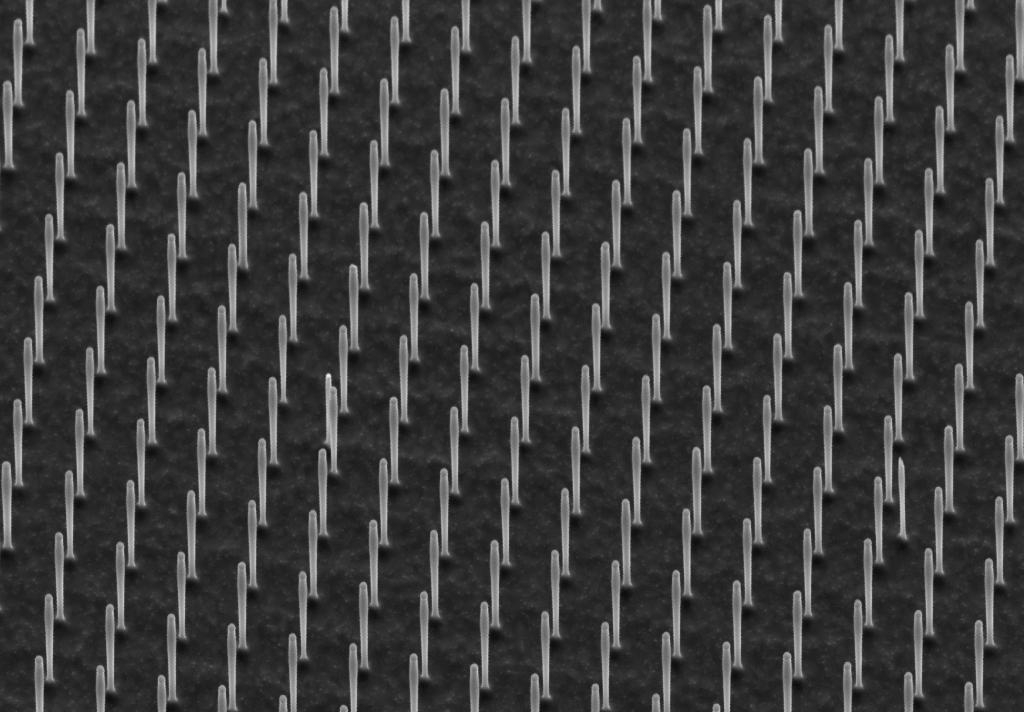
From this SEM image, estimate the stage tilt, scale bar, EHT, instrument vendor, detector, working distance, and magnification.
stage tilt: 45°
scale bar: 1000 nm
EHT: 3 kV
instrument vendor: Zeiss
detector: SE2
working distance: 10.8 mm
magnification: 6.33 K X